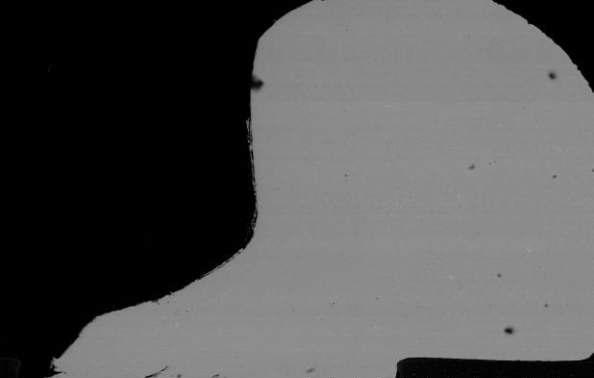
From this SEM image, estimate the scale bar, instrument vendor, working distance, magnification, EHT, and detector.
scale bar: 400000 nm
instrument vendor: Hitachi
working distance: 9.7 mm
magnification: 0.13 K X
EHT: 20 kV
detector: YAGBSE